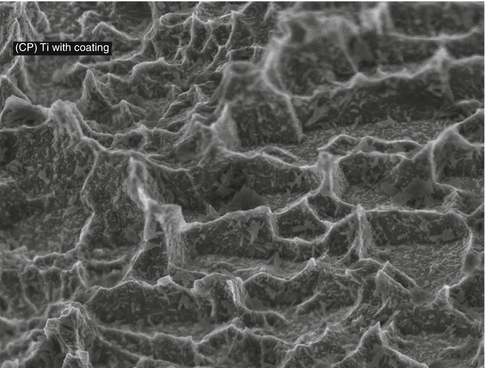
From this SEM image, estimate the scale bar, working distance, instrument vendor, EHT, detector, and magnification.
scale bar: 1000 nm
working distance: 3 mm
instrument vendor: JEOL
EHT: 3 kV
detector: SEI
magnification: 20 K X